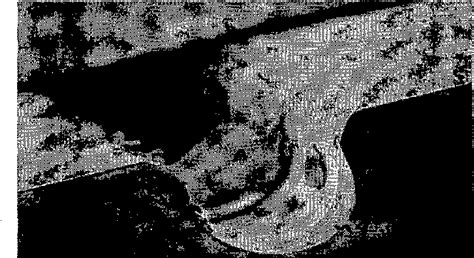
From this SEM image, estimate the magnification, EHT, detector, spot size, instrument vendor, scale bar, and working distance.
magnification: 0.481 K X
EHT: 2 kV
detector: SE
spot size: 2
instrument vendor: FEI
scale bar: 50000 nm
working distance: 10 mm